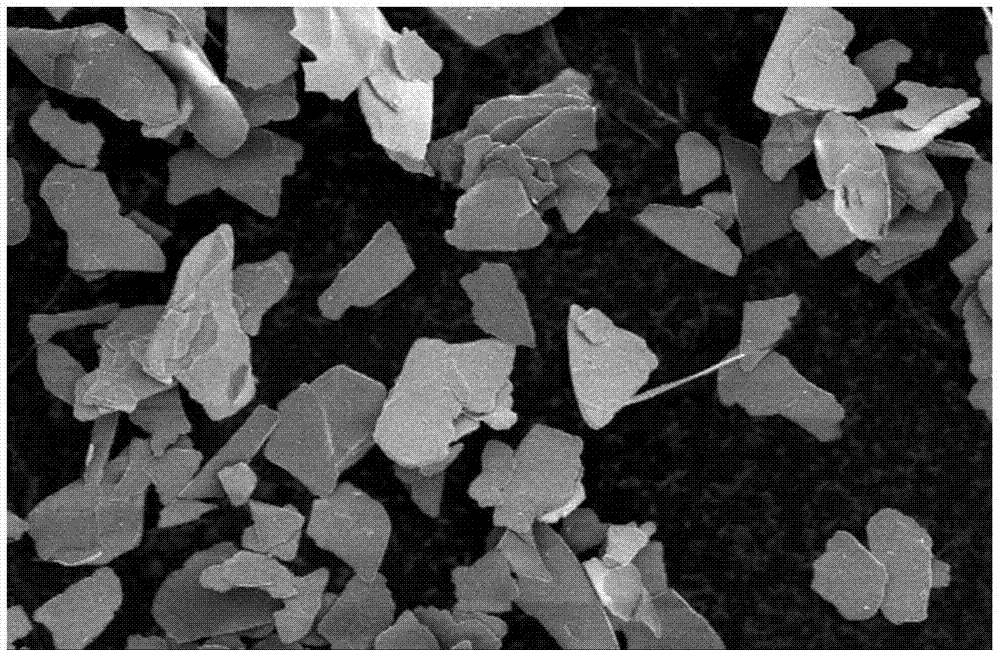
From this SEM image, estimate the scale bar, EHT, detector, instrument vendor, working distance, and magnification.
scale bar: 100000 nm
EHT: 5 kV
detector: SE(M)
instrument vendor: Hitachi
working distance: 5.2 mm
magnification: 0.5 K X